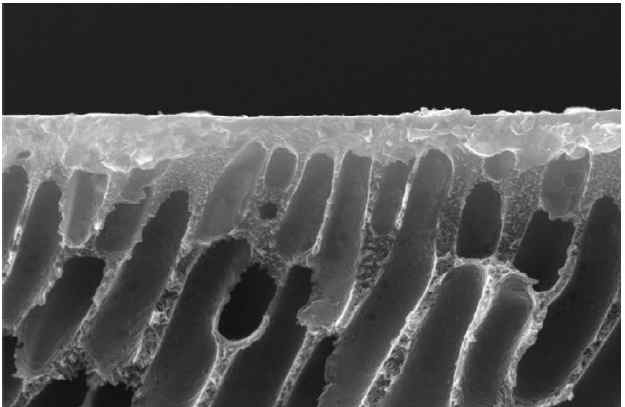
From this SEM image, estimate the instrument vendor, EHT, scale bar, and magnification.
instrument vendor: JEOL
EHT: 30 kV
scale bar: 10000 nm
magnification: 2.3 K X